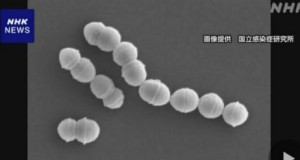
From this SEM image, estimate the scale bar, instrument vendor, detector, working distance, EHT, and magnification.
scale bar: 2000 nm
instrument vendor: Hitachi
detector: SE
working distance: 12.1 mm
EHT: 5 kV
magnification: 20 K X